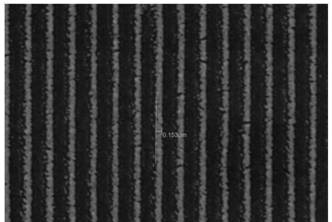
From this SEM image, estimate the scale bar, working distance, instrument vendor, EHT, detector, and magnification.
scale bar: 1000 nm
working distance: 10 mm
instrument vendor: JEOL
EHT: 15 kV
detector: SEI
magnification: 15 K X